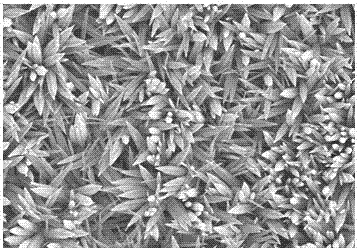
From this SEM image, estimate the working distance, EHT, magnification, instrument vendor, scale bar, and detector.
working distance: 10.9 mm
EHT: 3 kV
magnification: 10 K X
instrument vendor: Hitachi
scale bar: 5000 nm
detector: SE(M)